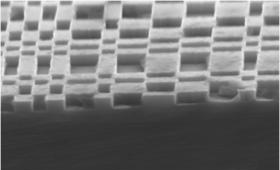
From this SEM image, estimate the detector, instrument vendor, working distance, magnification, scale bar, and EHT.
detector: InLens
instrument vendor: Zeiss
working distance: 5 mm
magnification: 50 K X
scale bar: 200 nm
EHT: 5 kV